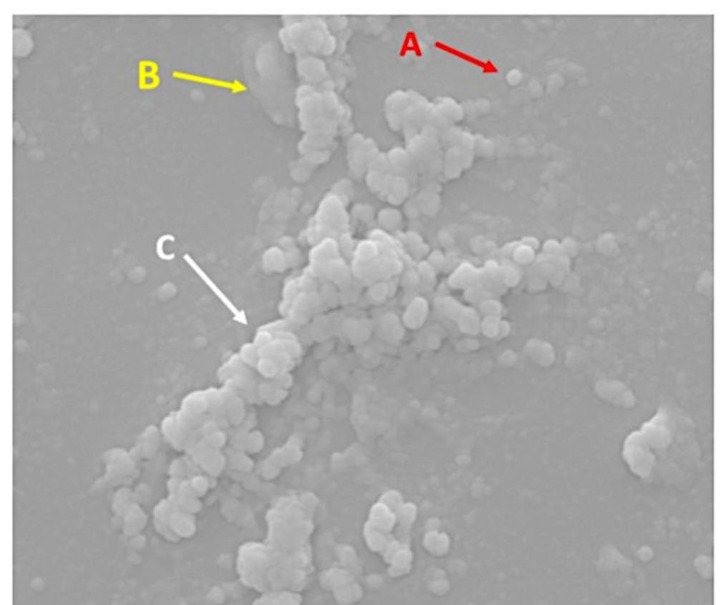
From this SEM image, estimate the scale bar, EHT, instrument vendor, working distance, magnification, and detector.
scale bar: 2000 nm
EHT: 25 kV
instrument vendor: FEI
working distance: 10.1 mm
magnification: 30 K X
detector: SE